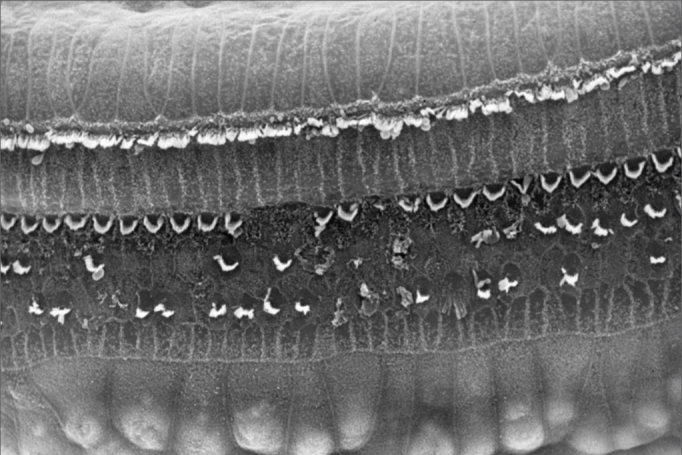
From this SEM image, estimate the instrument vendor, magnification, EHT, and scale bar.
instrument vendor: JEOL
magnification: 0.75 K X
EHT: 25 kV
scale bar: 10000 nm